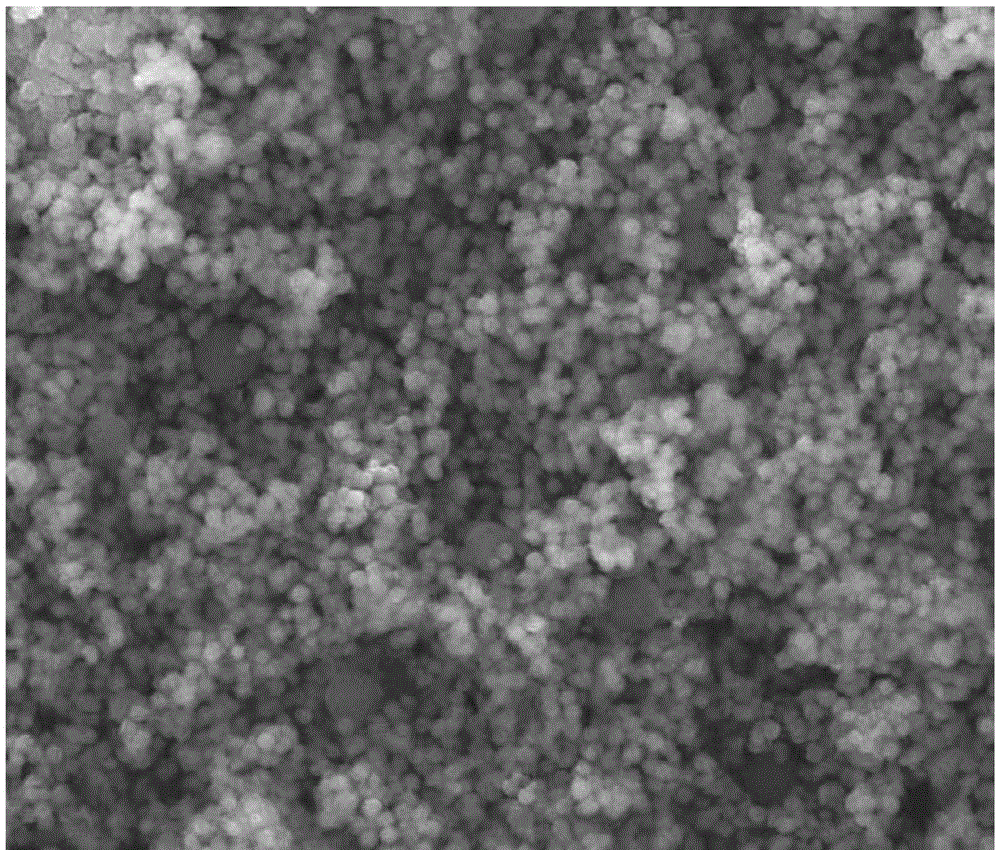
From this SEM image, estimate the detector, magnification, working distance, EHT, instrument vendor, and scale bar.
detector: SE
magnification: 20 K X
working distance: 10.4 mm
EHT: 30 kV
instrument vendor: FEI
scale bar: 5000 nm